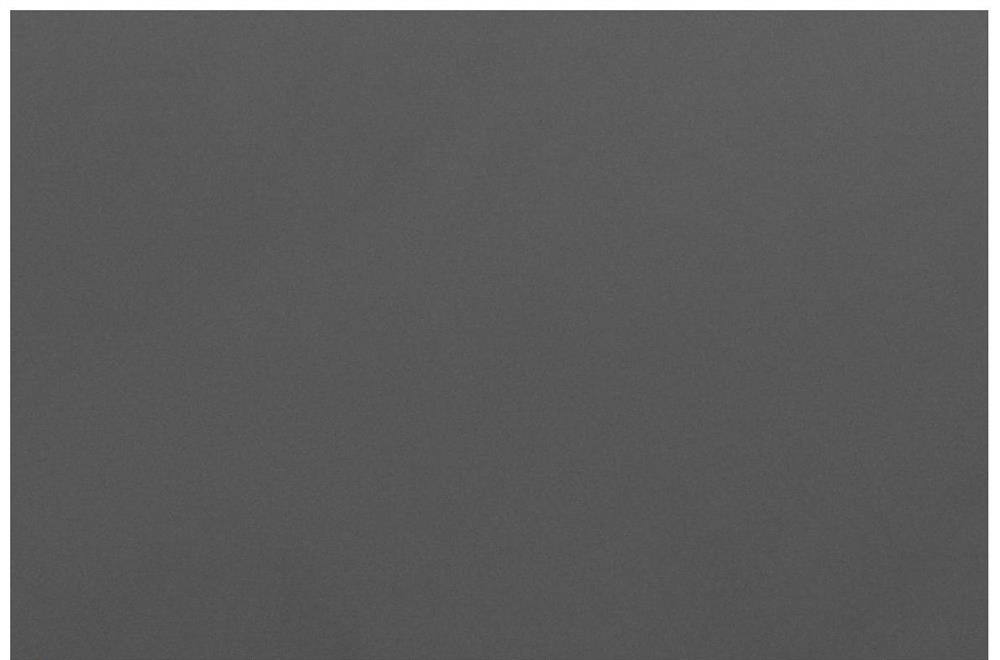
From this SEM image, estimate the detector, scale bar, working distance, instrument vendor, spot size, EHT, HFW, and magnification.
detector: ETD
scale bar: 10000 nm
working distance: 11.8 mm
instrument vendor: Thermo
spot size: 2.5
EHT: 10 kV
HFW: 41.4 µm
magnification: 5 K X